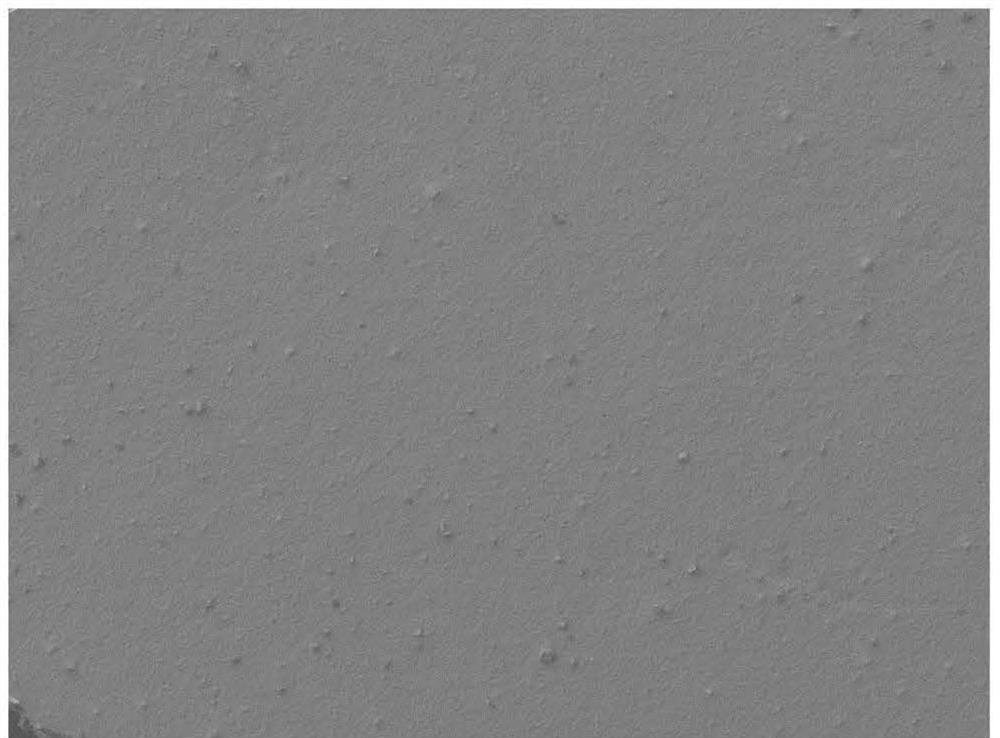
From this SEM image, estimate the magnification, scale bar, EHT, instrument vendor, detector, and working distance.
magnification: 0.1 K X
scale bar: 100000 nm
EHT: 5 kV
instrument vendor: JEOL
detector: SEI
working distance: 8 mm